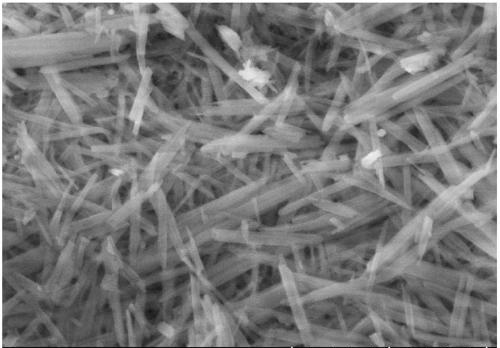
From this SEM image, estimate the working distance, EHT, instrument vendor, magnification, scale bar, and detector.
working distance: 4.3 mm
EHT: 5 kV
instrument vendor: Hitachi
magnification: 100 K X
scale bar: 500 nm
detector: SE(U)